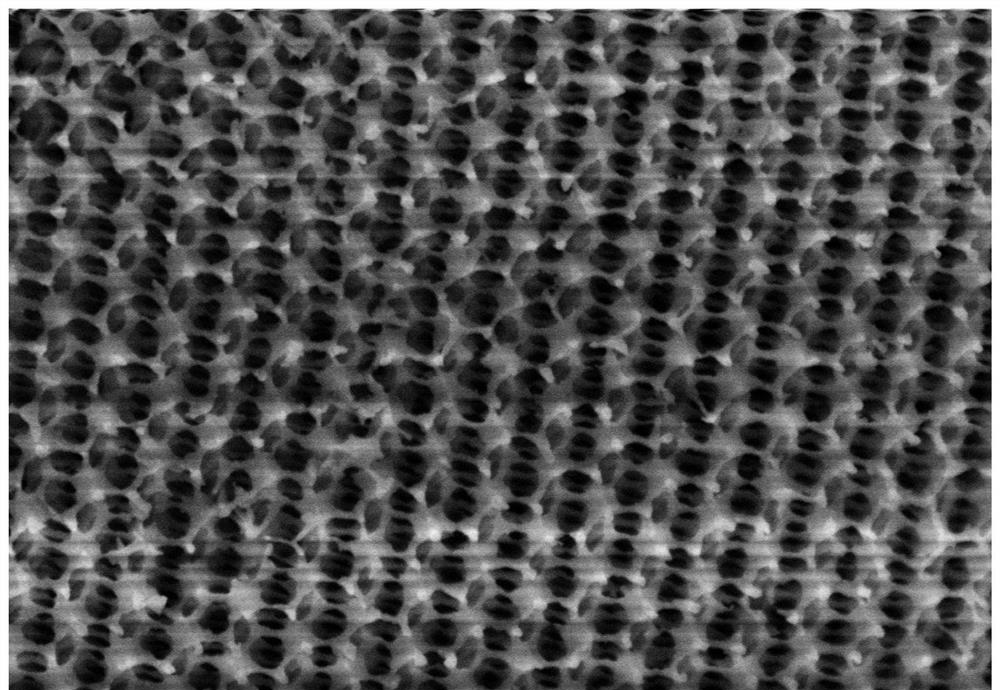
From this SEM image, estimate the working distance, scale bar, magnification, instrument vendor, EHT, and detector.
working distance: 8.8 mm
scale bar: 1000 nm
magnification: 50 K X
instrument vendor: Hitachi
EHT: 5 kV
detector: SE(U)